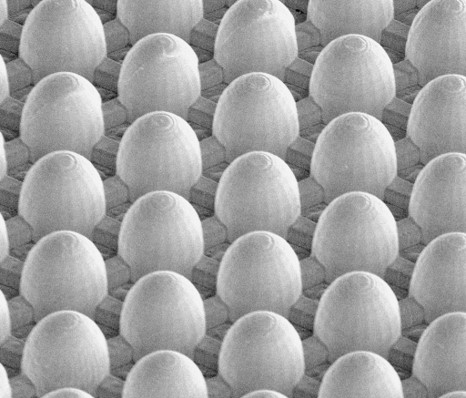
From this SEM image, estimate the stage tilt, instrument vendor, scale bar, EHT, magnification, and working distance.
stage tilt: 0°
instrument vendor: FEI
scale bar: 50000 nm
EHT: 5 kV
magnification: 0.696 K X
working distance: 10.5 mm